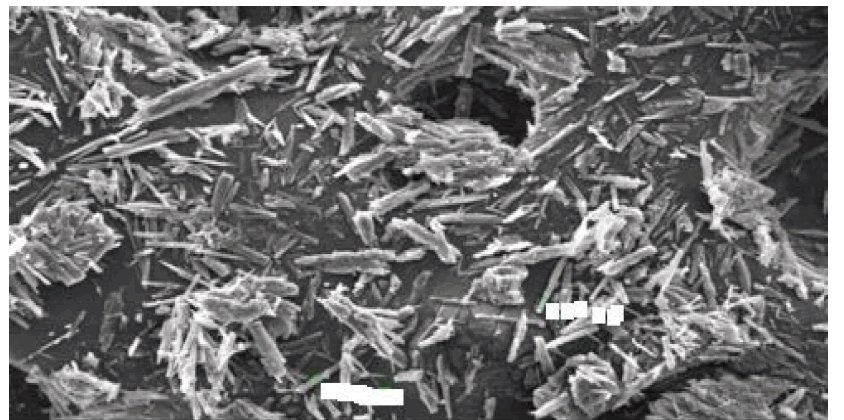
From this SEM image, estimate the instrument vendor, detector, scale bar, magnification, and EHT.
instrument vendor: JEOL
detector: SEI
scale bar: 100000 nm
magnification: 0.25 K X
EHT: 20 kV